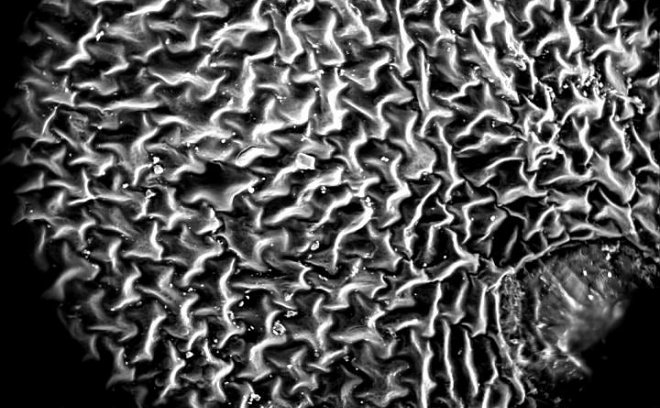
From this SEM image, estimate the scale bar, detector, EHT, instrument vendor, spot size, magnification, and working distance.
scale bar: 300000 nm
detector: GSED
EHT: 15 kV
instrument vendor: FEI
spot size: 5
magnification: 0.4 K X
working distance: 7.9 mm